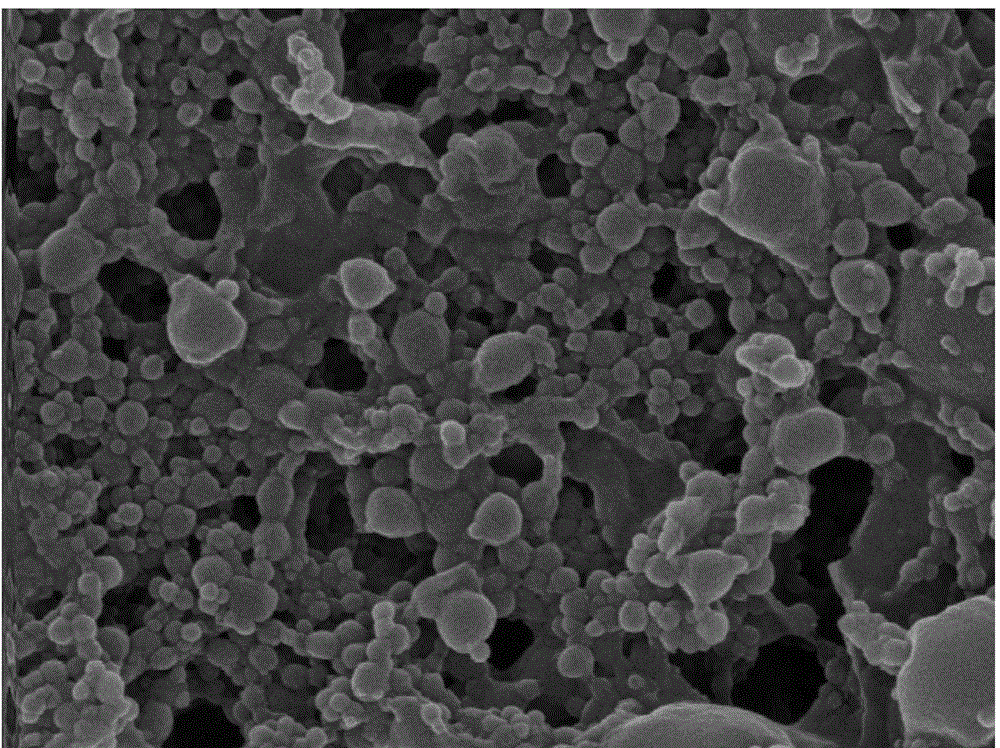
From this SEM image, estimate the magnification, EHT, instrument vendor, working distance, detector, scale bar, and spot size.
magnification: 28.643 K X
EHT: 10 kV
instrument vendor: FEI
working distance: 5.5 mm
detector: TLD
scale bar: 1000 nm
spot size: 4